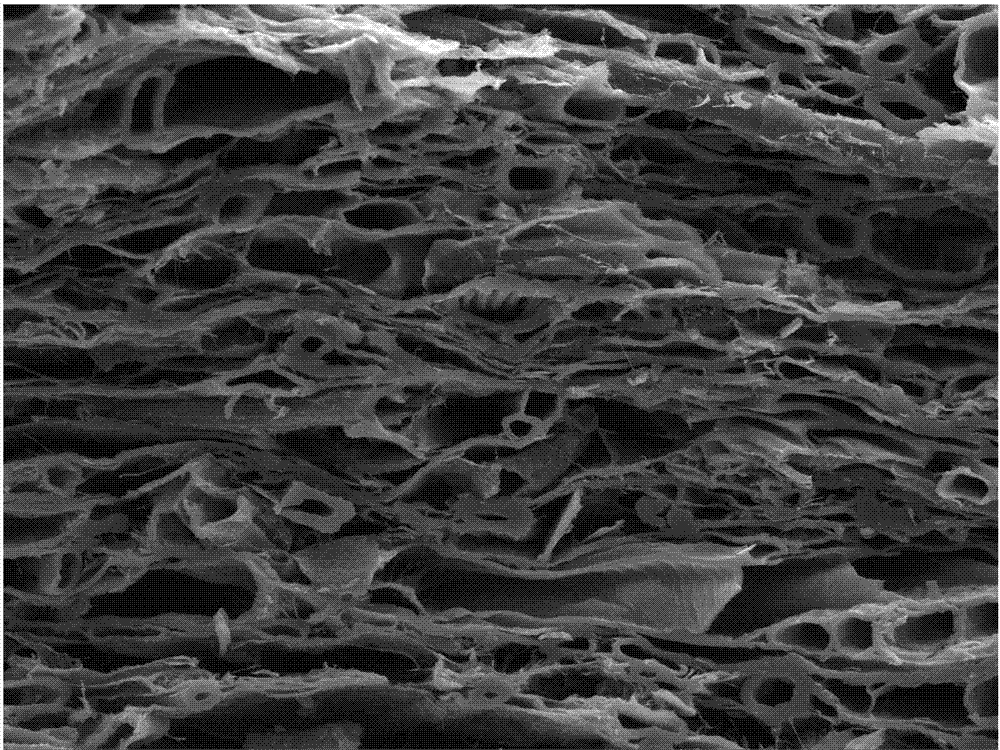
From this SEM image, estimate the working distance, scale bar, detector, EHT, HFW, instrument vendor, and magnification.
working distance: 8.39 mm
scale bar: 50000 nm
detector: SE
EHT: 20 kV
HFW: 277 µm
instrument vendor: Tescan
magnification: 1 K X